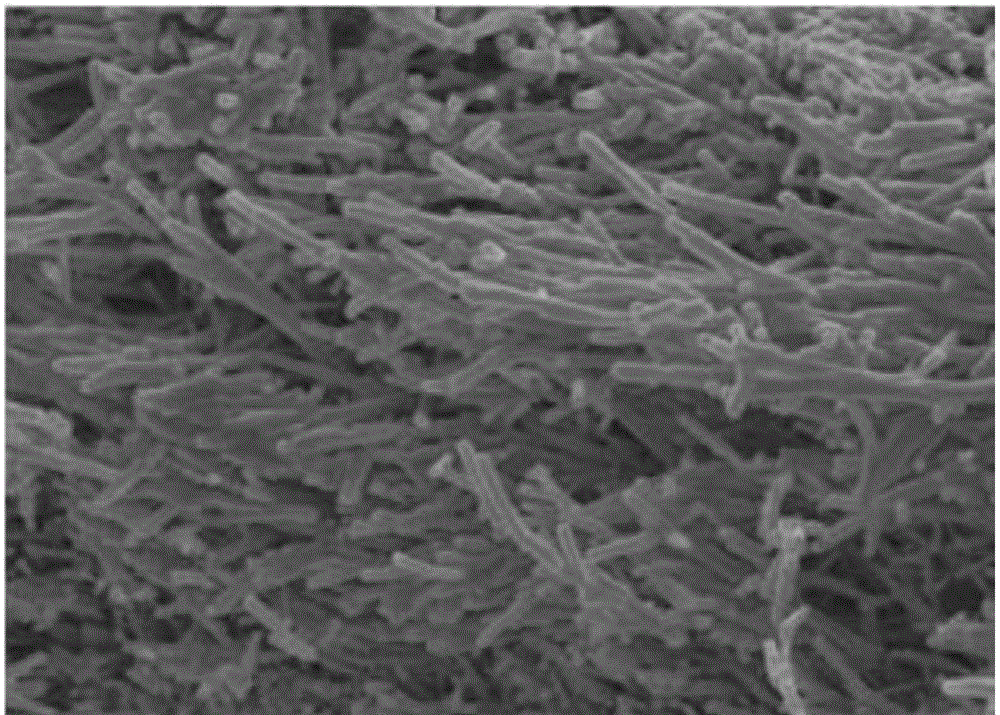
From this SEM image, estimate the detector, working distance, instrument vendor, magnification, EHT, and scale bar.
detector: SE(U)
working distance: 9.4 mm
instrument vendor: Hitachi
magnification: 10 K X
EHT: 5 kV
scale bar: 5000 nm